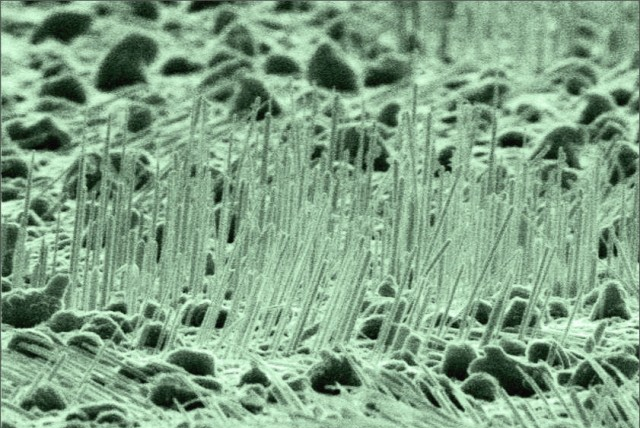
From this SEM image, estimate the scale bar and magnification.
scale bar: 1000 nm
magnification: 20 K X